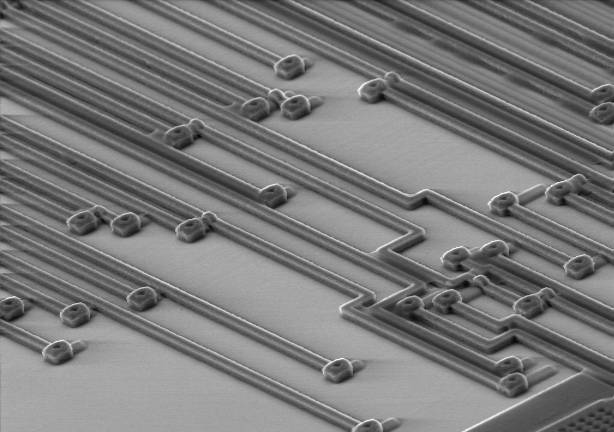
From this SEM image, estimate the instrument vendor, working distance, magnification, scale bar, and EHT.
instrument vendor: JEOL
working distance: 24 mm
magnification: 1 K X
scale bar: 20000 nm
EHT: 10 kV